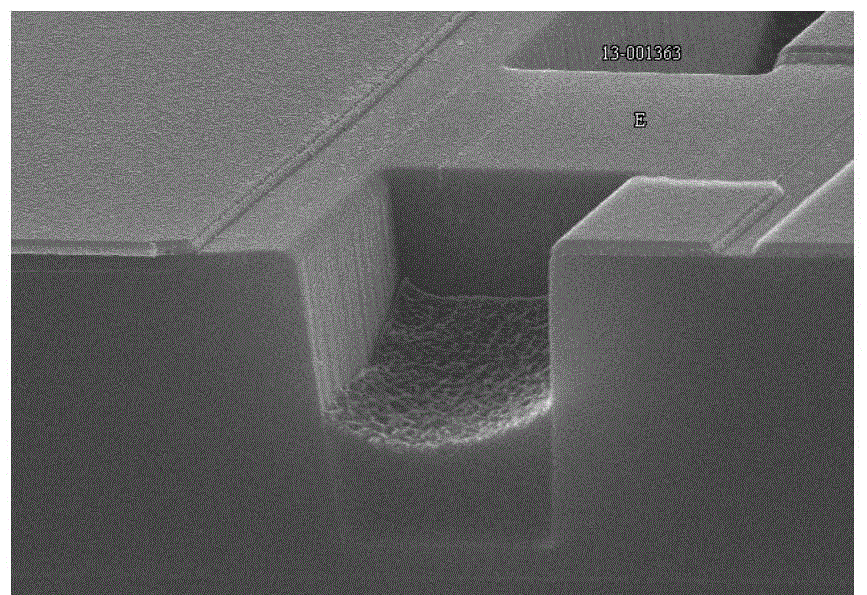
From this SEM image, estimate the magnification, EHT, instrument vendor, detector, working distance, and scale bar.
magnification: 15 K X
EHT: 15 kV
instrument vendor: Hitachi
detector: SE(U)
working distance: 7.5 mm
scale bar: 3000 nm